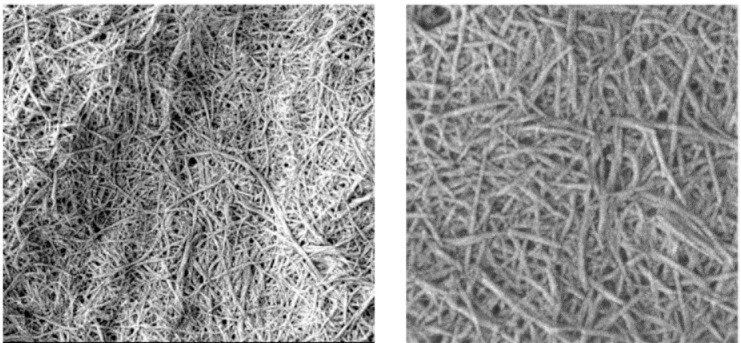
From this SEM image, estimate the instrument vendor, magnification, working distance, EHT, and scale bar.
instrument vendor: JEOL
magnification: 30 K X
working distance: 7.9 mm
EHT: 1.5 kV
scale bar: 200 nm